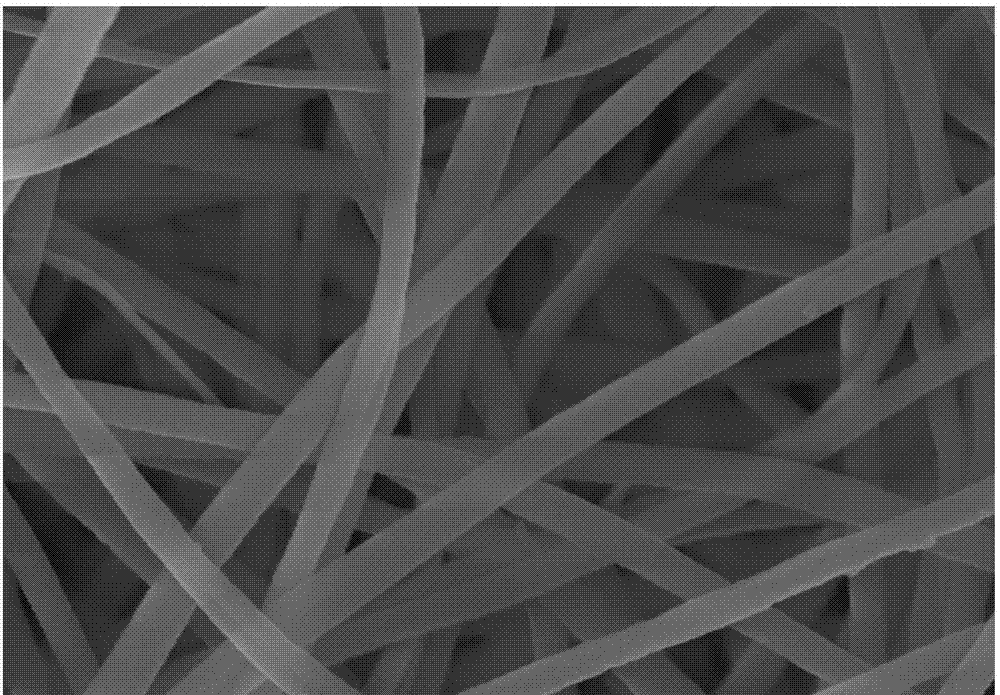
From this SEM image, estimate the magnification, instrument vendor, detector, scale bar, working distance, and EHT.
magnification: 20 K X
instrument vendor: Hitachi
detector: SE(M)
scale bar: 2000 nm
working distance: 17.7 mm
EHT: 10 kV